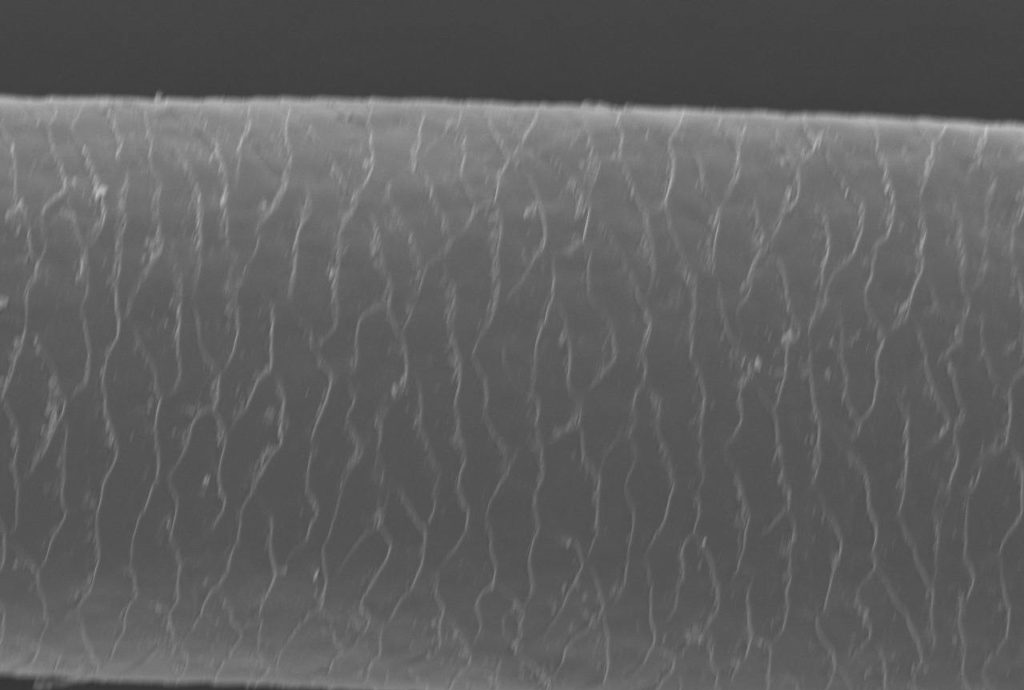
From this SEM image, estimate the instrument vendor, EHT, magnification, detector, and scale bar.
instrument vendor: JEOL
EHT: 10 kV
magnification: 0.24 K X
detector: SEI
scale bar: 100000 nm